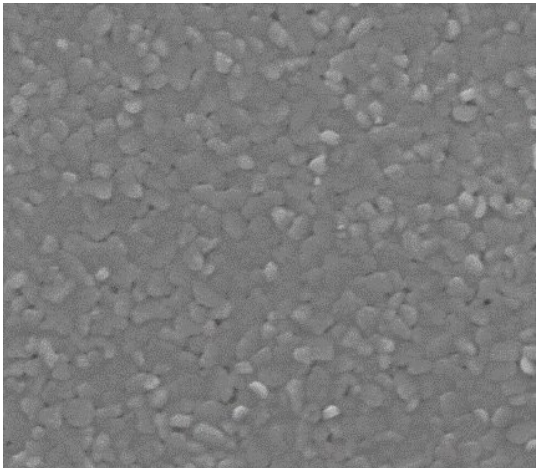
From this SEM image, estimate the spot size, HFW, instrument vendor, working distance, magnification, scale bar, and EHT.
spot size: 3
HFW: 2.11 µm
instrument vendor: FEI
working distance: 9.9 mm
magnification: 60.763 K X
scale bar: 1000 nm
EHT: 10 kV